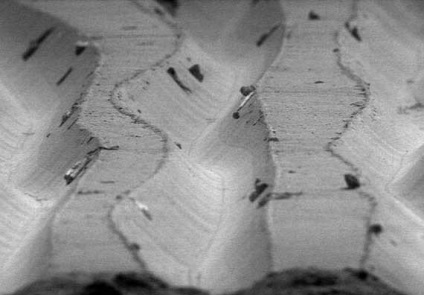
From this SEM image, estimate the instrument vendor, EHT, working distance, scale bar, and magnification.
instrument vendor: JEOL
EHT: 5 kV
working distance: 27 mm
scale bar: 50000 nm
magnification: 0.5 K X